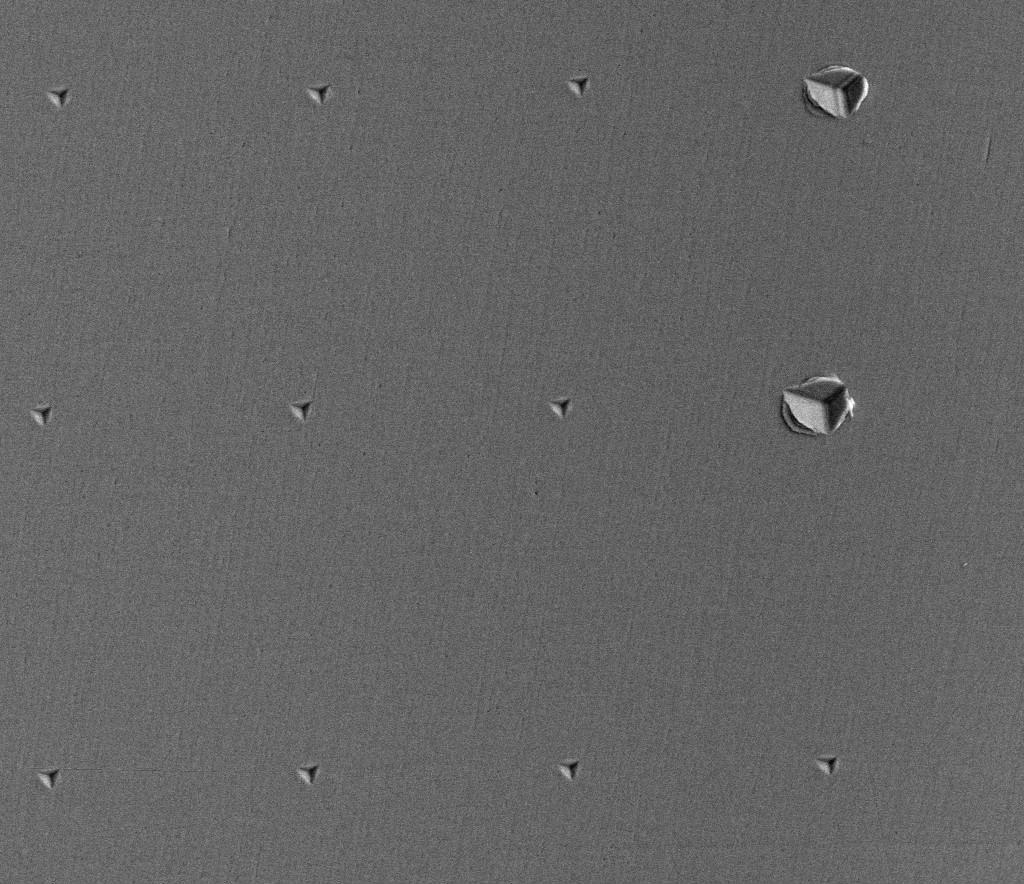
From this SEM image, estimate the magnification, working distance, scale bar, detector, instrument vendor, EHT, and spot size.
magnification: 1.25 K X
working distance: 12 mm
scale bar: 50000 nm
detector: ETD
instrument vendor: FEI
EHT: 15 kV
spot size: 5